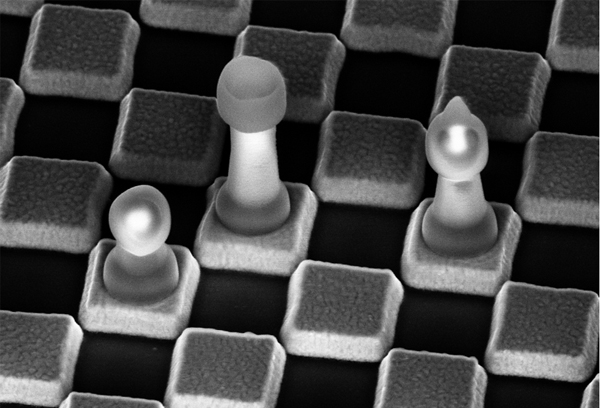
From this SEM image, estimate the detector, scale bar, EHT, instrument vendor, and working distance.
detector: InLens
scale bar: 200 nm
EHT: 5 kV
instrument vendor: Zeiss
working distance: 10 mm